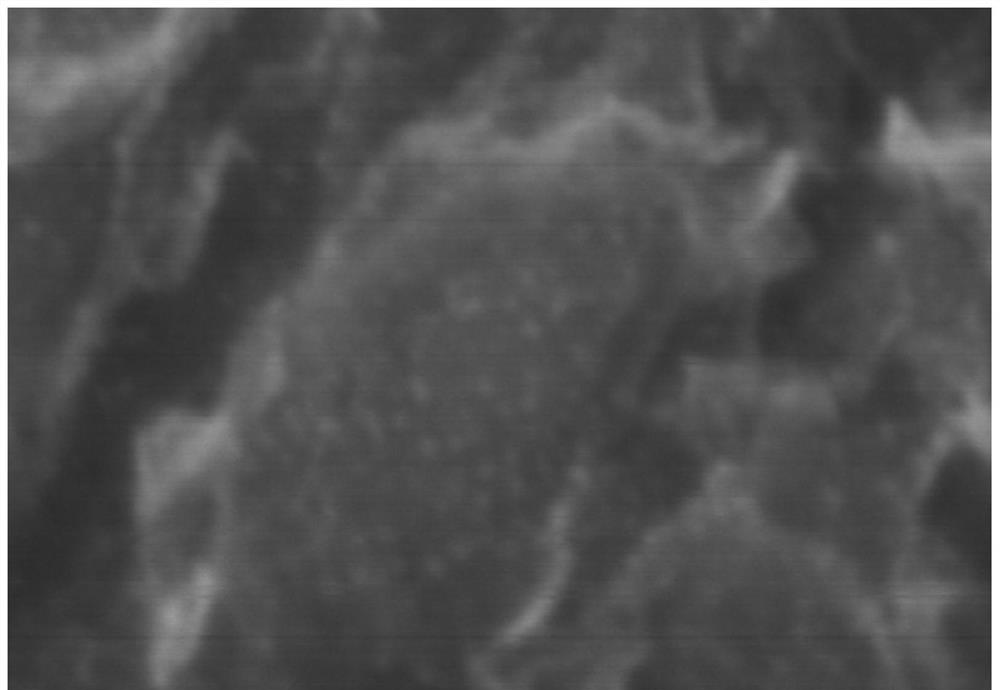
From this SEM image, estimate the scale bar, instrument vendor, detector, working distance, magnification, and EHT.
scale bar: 100 nm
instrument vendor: Hitachi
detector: SE(U)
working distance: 8.8 mm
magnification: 500 K X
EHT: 5 kV